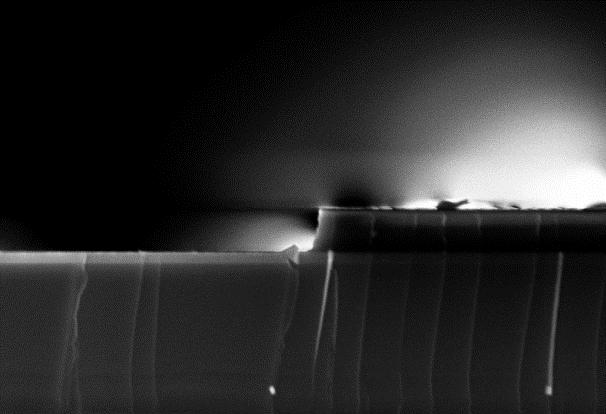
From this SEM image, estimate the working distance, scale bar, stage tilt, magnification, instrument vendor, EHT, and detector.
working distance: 5.1 mm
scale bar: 200 nm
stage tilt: -1.7°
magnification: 50 K X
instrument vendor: Zeiss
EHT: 5 kV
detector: InLens